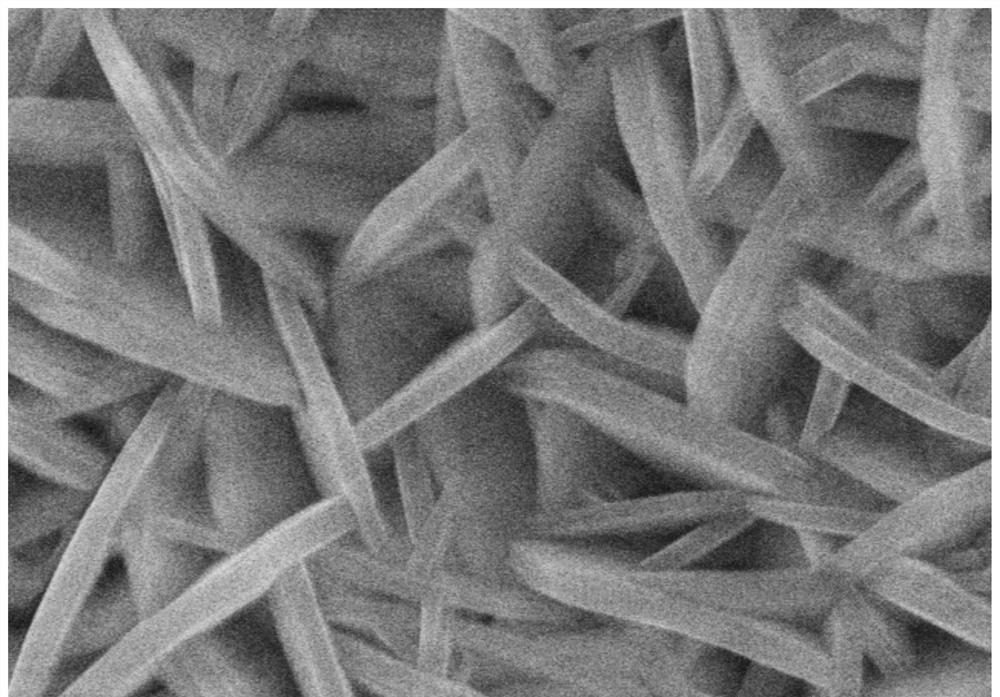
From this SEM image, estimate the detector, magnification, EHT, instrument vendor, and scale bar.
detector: SE(U)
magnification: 50 K X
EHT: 15 kV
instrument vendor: Hitachi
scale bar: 1000 nm